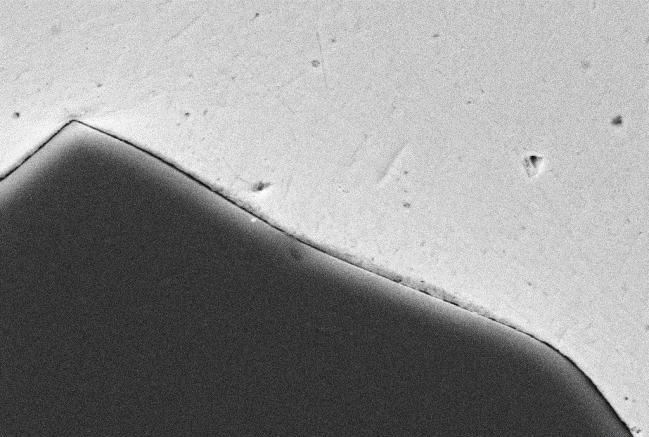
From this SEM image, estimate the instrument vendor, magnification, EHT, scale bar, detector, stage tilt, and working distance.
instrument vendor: Zeiss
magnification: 2 K X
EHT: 20 kV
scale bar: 2000 nm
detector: SE2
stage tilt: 0°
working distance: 16.4 mm